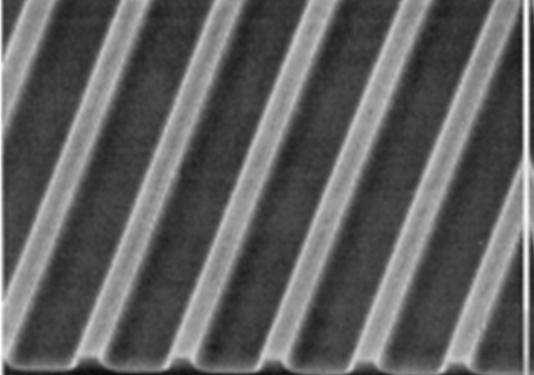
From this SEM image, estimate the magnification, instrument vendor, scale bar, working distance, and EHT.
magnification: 5 K X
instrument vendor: JEOL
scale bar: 1000 nm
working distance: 39 mm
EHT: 15 kV